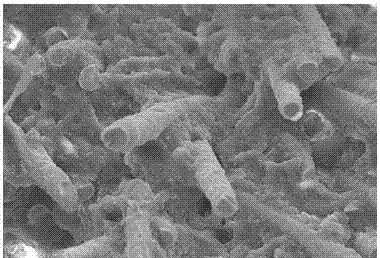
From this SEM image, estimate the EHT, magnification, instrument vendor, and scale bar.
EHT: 20 kV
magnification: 0.5 K X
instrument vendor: JEOL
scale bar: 50000 nm